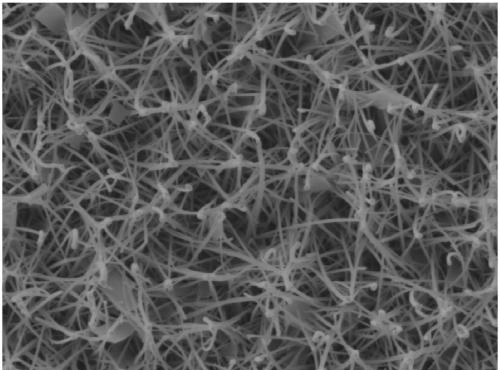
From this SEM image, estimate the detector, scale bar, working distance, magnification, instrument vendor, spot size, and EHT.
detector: SED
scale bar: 5000 nm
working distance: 10.7 mm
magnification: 5 K X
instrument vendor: JEOL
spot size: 30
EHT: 10 kV